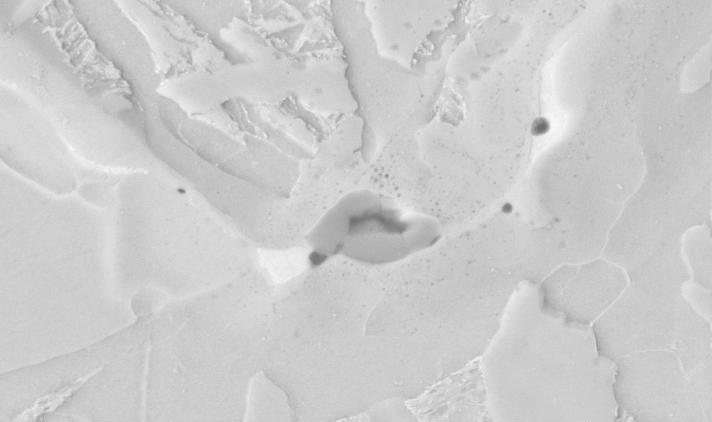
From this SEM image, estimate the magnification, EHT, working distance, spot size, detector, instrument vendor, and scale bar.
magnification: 5 K X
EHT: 20 kV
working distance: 5.2 mm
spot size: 3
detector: BSE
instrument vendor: FEI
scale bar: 5000 nm